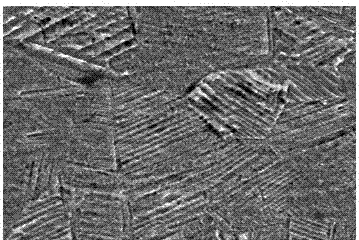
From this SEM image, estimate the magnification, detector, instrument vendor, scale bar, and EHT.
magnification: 2.5 K X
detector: SEI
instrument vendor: JEOL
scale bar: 10000 nm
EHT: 20 kV